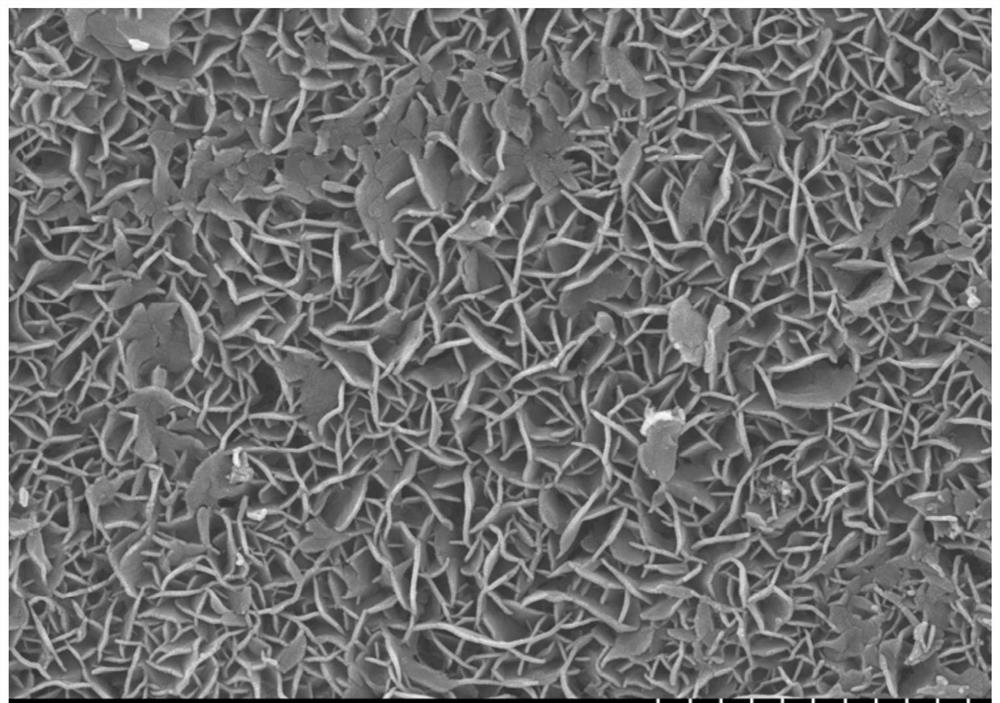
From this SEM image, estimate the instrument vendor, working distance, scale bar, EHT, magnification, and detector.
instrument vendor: Hitachi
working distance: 5.6 mm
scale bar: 2000 nm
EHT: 15 kV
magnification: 20 K X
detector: SE(L)